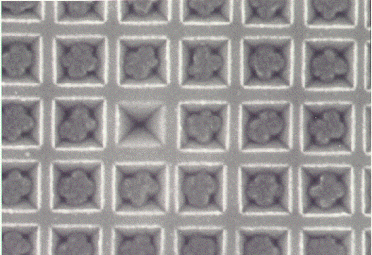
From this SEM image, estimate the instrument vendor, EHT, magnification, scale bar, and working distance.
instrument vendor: JEOL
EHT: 5 kV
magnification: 100 K X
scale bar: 200 nm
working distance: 3 mm